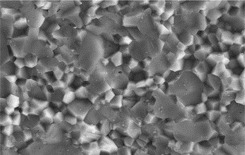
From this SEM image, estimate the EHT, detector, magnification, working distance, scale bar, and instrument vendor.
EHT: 20 kV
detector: SE2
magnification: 2.2 K X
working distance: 7.9 mm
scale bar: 2000 nm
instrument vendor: Zeiss